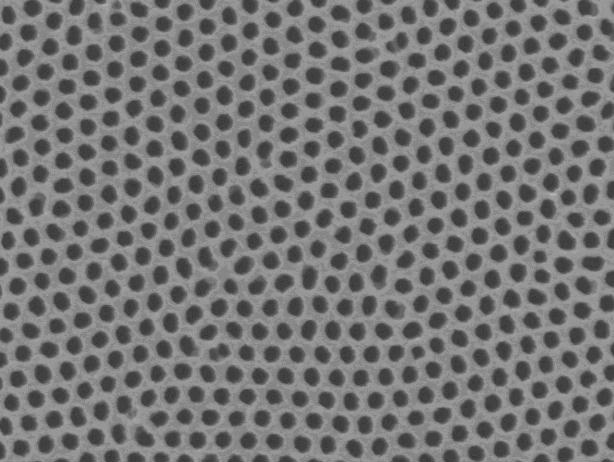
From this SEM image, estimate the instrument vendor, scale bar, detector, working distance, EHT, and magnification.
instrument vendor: JEOL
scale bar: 100 nm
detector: SEI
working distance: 11.6 mm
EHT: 15 kV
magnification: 50 K X